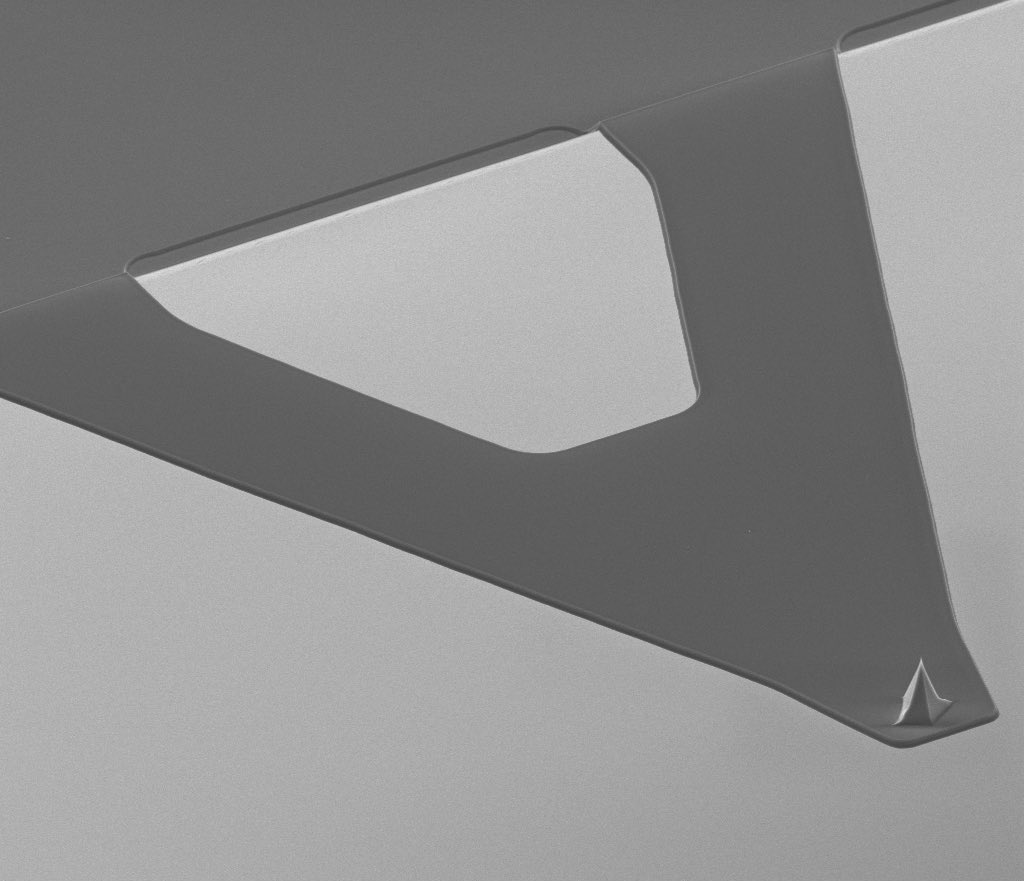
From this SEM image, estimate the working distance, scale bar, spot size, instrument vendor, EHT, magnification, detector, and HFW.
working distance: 11.2 mm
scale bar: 20000 nm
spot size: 2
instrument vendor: FEI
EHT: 5 kV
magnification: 4 K X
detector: ETD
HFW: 74.6 µm